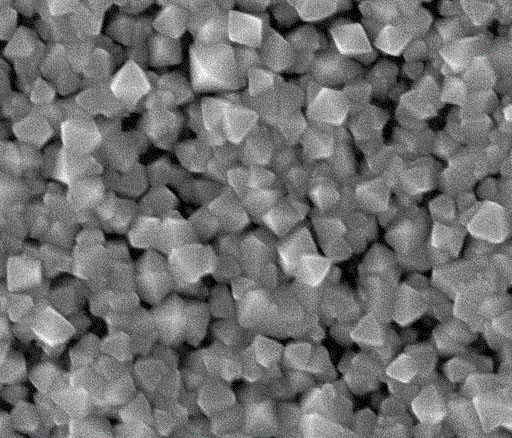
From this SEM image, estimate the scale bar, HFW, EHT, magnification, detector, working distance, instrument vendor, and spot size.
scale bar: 400 nm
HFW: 1.49 µm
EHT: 10 kV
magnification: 100 K X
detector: ETD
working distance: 4.7 mm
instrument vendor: FEI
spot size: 2